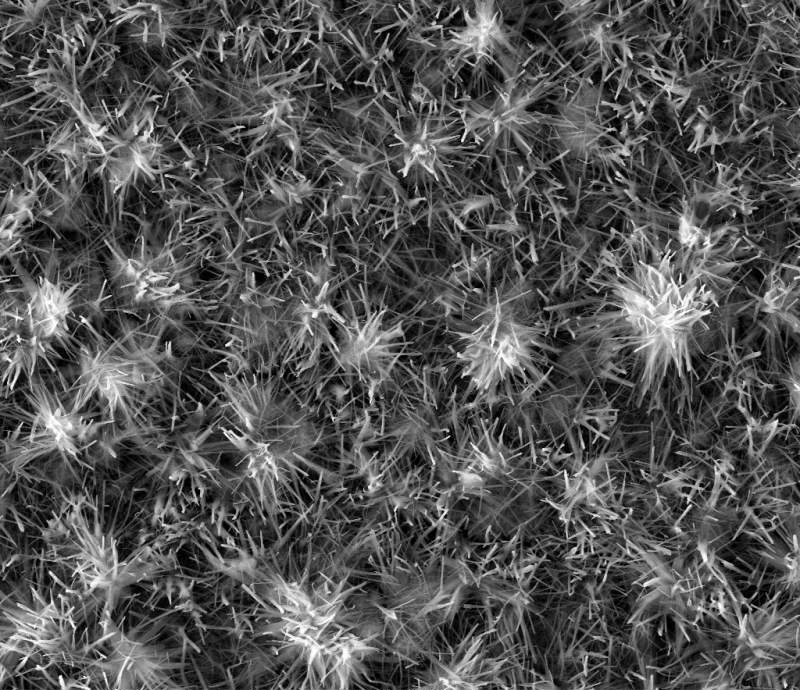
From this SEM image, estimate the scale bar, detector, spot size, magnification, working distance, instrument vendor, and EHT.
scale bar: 20000 nm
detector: ETD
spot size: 3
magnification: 5 K X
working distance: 22.6 mm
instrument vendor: FEI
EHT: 20 kV